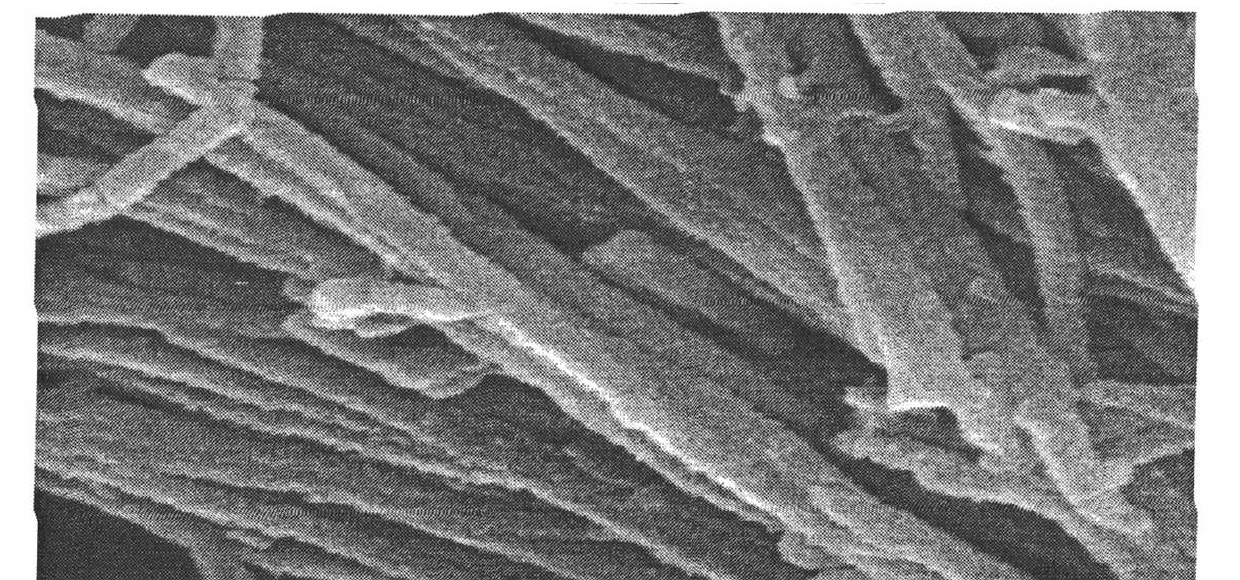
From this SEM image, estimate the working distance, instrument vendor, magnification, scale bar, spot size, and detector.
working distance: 4 mm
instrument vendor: FEI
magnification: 20 K X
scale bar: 1000 nm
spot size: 2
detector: SE